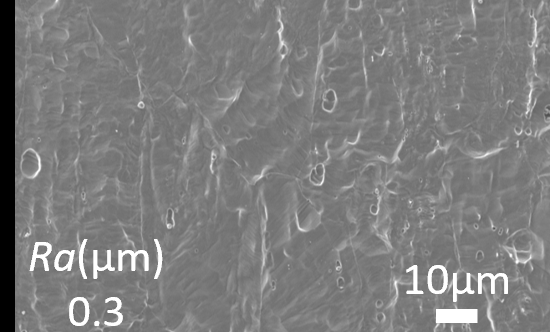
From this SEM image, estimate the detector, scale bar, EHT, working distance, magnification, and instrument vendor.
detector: SED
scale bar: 10000 nm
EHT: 10 kV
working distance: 12.1 mm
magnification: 1 K X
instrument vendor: JEOL